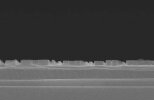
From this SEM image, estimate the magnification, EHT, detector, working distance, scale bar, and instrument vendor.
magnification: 10 K X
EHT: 15 kV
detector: SE(M)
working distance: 15.8 mm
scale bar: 3000 nm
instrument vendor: Hitachi